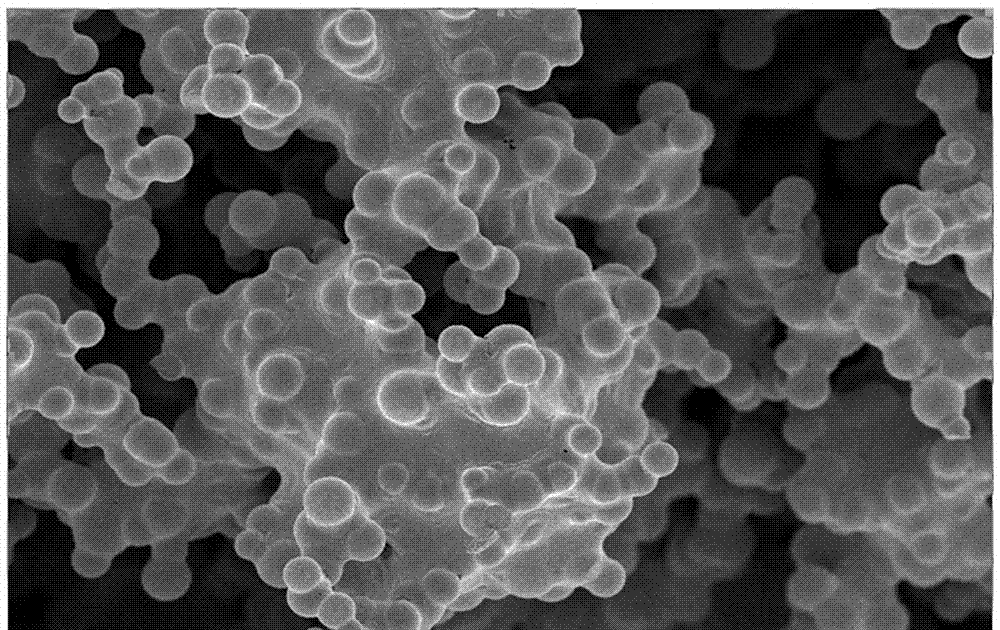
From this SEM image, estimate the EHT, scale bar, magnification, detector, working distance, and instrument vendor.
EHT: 5 kV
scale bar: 10000 nm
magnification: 1 K X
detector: InLens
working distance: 4.6 mm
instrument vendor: Zeiss